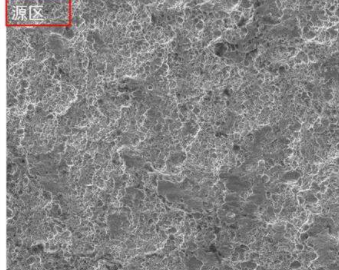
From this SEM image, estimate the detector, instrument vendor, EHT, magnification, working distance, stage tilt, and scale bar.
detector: ETD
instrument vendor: FEI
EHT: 15 kV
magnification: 1 K X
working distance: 16.5 mm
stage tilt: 0°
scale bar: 100000 nm